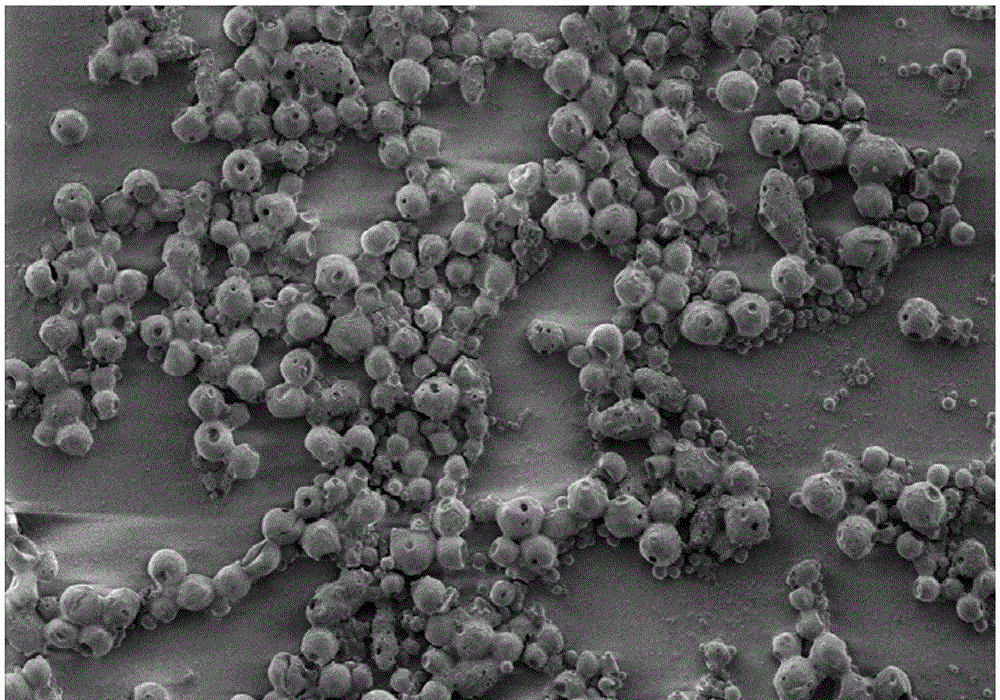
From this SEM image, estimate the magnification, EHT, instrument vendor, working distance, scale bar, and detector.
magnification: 0.1 K X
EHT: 1 kV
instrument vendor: Hitachi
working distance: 8.6 mm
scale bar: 500000 nm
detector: SE(M)